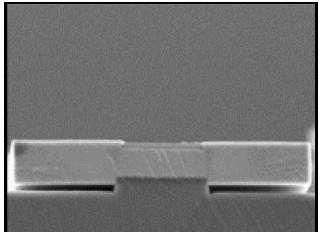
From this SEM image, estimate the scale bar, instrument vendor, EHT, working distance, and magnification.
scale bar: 1000 nm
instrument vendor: JEOL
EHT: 5 kV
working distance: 16 mm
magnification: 15 K X